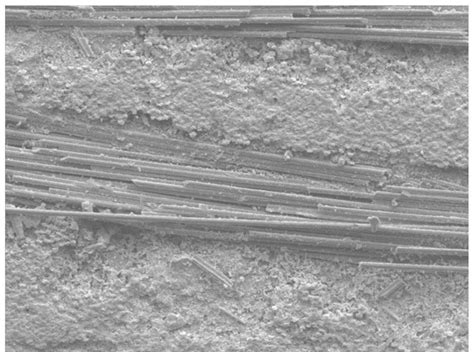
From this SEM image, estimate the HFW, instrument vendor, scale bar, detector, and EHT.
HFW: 600 µm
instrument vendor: JEOL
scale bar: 100000 nm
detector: SEI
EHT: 15 kV